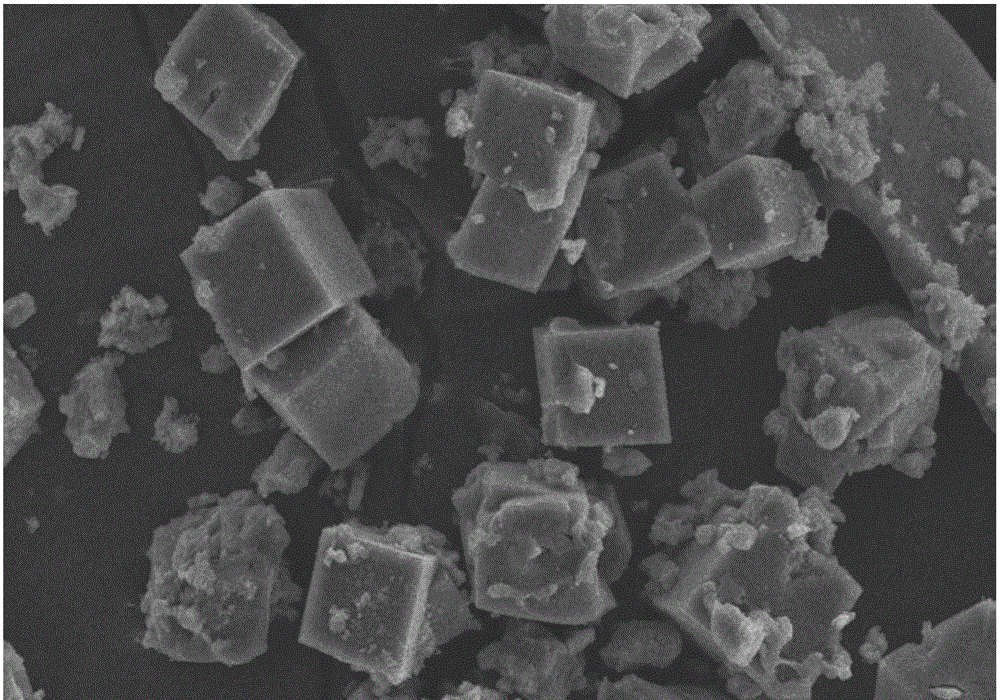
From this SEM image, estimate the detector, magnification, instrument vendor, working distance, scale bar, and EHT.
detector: SE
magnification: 1 K X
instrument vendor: Hitachi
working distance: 6 mm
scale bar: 50000 nm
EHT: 15 kV